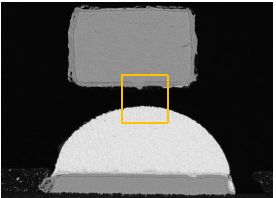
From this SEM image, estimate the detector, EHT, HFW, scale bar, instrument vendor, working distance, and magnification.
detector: BSE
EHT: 20 kV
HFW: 444 µm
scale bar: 100000 nm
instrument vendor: Tescan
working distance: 14.9 mm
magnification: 0.624 K X